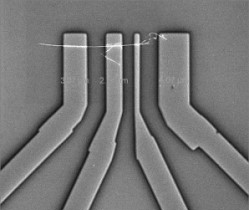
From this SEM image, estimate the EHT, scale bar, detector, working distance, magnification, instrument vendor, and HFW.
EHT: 15 kV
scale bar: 10000 nm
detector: ETD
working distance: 4 mm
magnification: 3.5 K X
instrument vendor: FEI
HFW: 36.6 µm